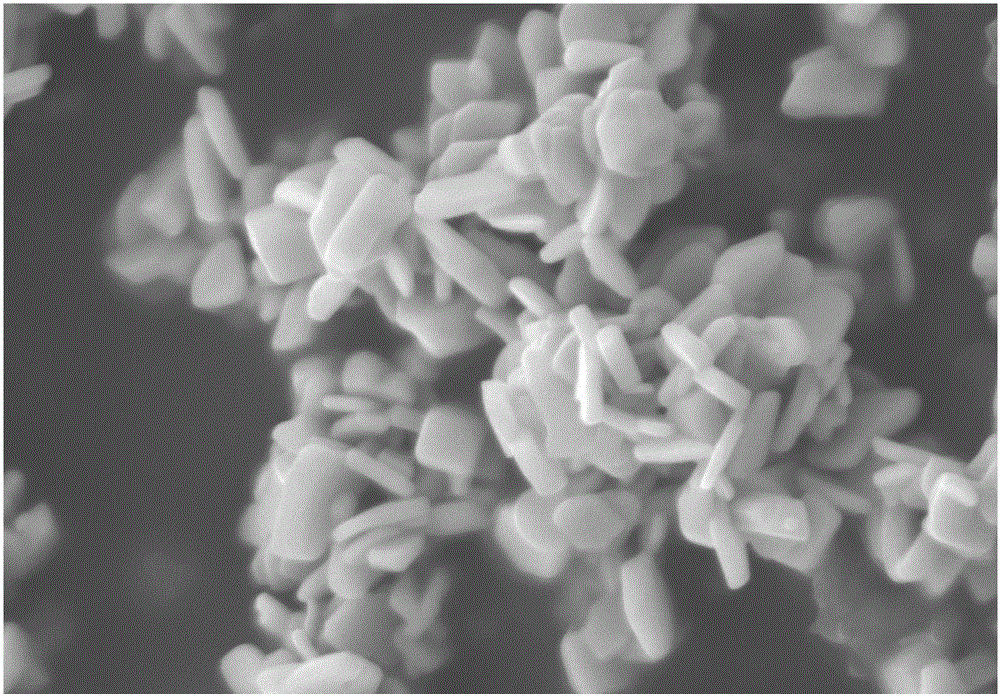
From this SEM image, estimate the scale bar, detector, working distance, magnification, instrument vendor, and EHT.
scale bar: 200 nm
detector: SE2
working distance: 9.8 mm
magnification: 40 K X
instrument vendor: Zeiss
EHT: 5 kV